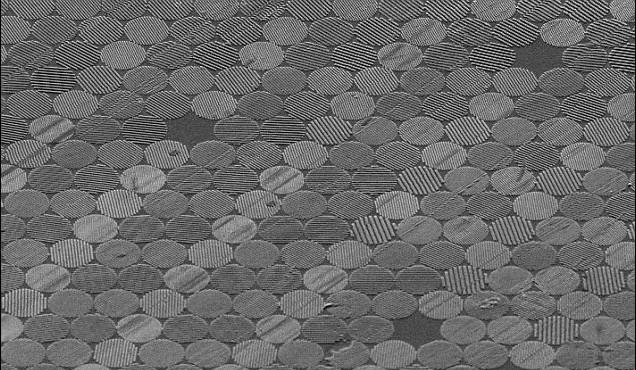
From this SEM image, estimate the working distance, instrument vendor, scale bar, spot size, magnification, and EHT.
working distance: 13 mm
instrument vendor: FEI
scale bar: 20000 nm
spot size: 3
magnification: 2 K X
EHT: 5 kV